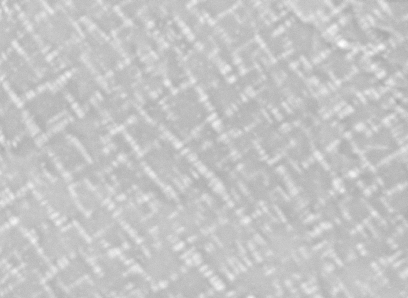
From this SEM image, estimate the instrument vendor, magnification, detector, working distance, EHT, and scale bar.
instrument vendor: JEOL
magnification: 3 K X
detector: COMP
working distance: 11 mm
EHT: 20 kV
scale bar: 1000 nm